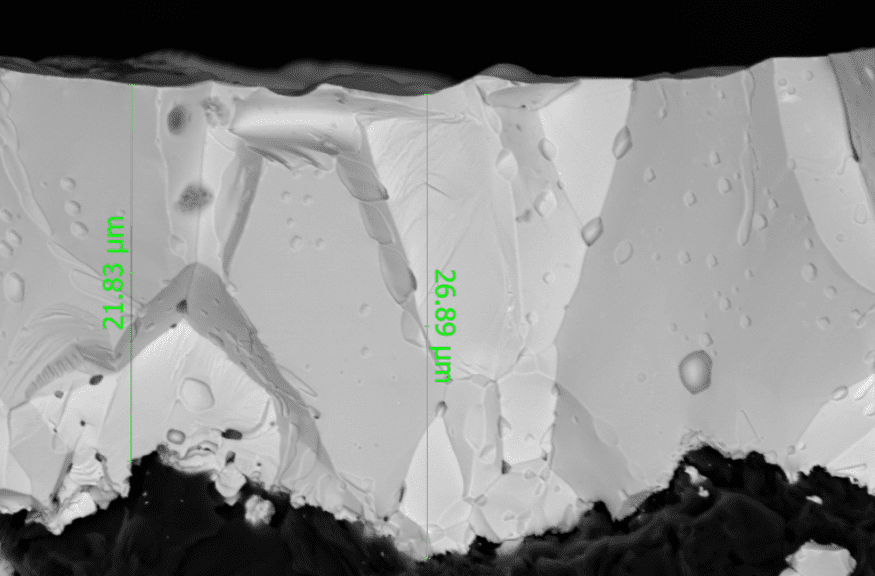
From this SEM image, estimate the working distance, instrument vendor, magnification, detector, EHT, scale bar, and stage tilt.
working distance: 7.4 mm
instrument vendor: Thermo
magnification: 2.5 K X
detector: ABS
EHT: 10 kV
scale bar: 10000 nm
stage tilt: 0°